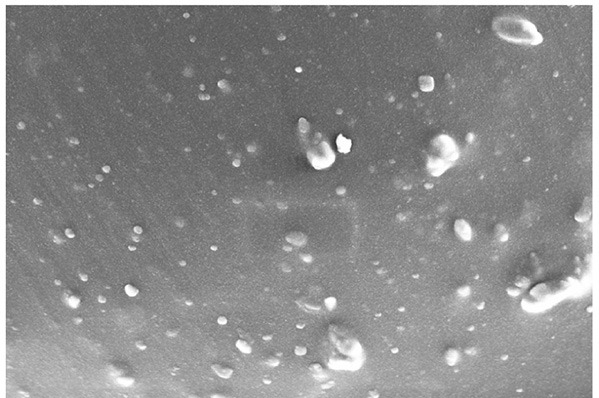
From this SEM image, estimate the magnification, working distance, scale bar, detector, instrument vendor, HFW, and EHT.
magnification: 20 K X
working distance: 2.8 mm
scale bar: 1000 nm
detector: InLens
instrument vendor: Zeiss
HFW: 18 µm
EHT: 5 kV